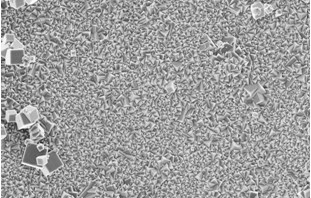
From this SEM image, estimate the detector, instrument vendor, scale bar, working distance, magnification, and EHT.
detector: InLens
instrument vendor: Zeiss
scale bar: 2000 nm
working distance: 7.6 mm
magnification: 5 K X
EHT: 5 kV